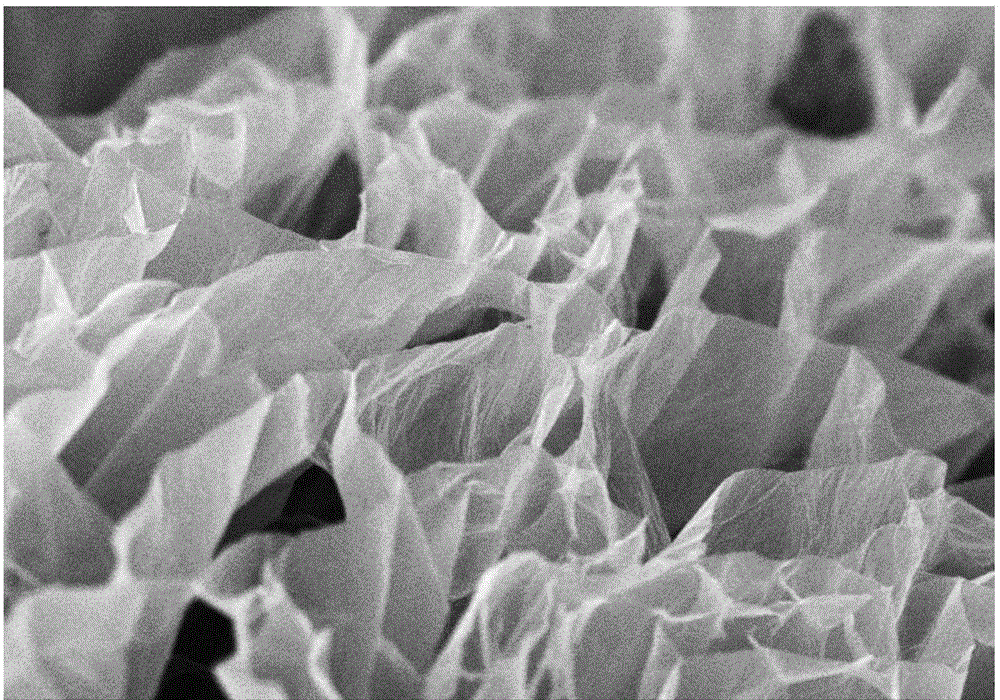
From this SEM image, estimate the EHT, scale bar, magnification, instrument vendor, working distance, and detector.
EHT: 3 kV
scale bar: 10000 nm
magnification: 5 K X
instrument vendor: Hitachi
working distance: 10.5 mm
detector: SE(M)